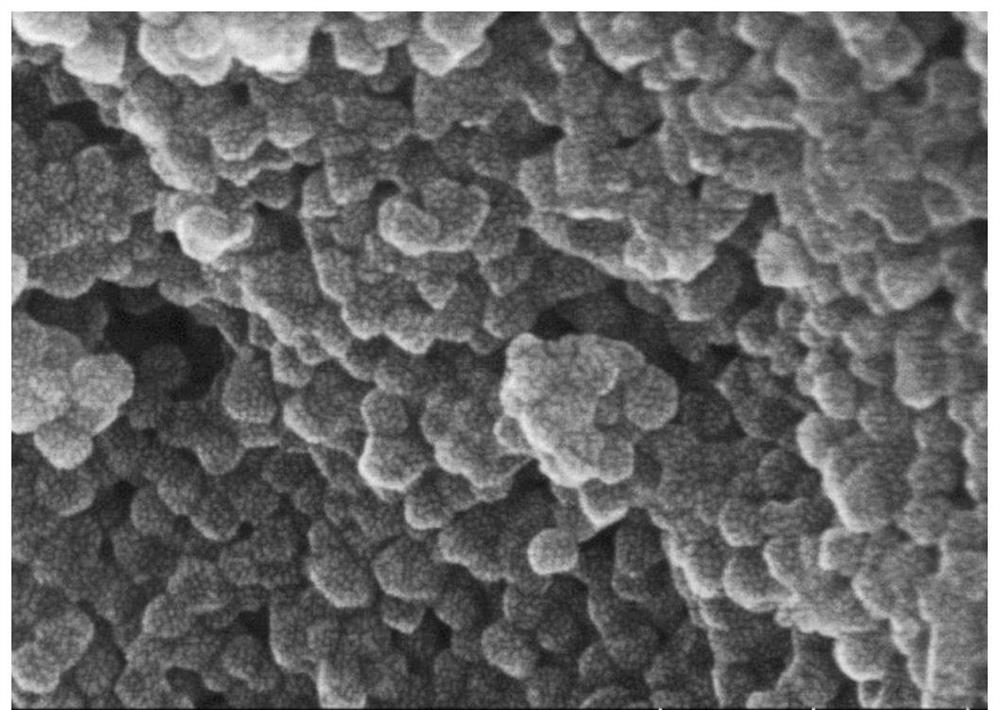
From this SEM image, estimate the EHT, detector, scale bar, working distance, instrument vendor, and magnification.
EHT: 10 kV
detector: SE(UL)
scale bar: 200 nm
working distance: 5.4 mm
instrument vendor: Hitachi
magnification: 200 K X